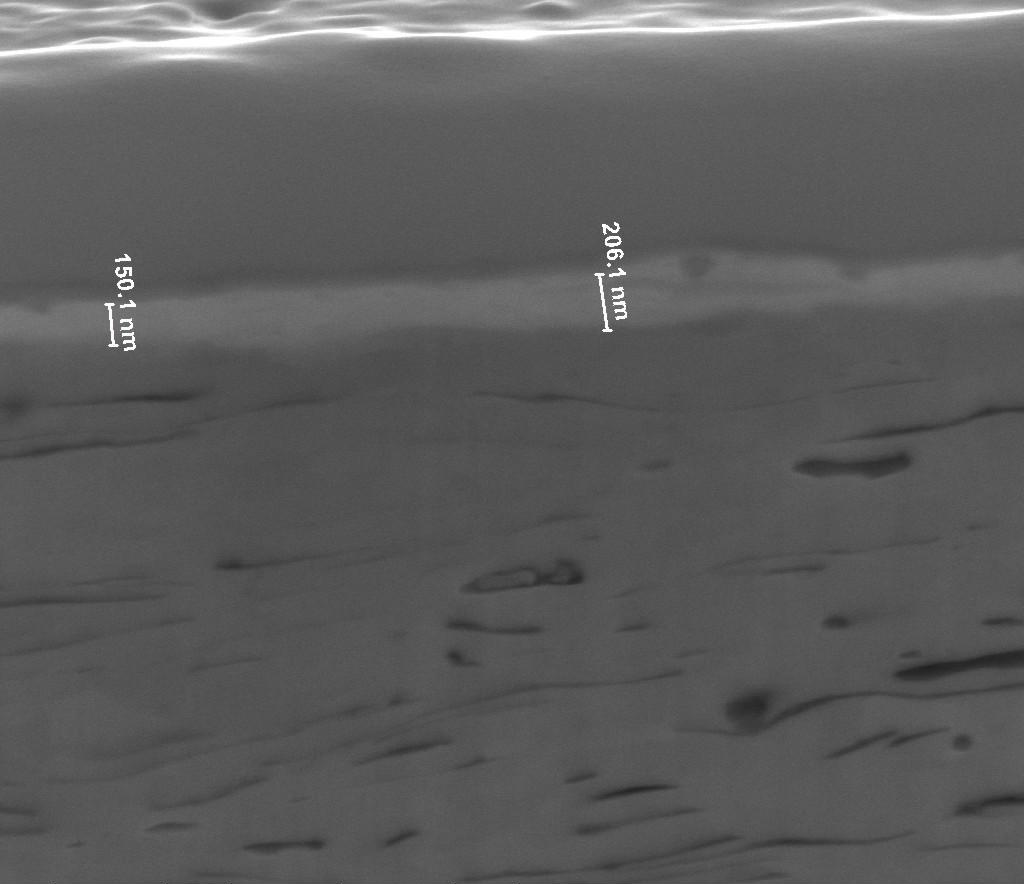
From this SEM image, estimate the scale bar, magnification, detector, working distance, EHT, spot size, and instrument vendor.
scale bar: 1000 nm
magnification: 40 K X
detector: ETD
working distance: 10.1 mm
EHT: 20 kV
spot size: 4.5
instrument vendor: FEI